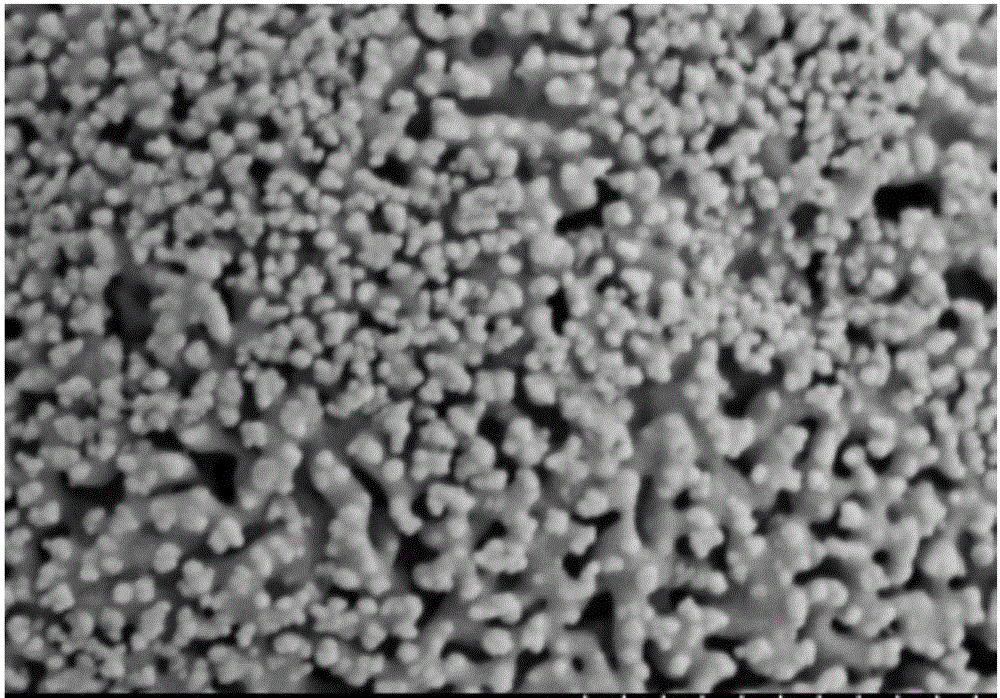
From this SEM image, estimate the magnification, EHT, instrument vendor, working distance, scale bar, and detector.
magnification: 50 K X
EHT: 3 kV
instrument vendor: Hitachi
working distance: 9.7 mm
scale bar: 1000 nm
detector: SE(M)